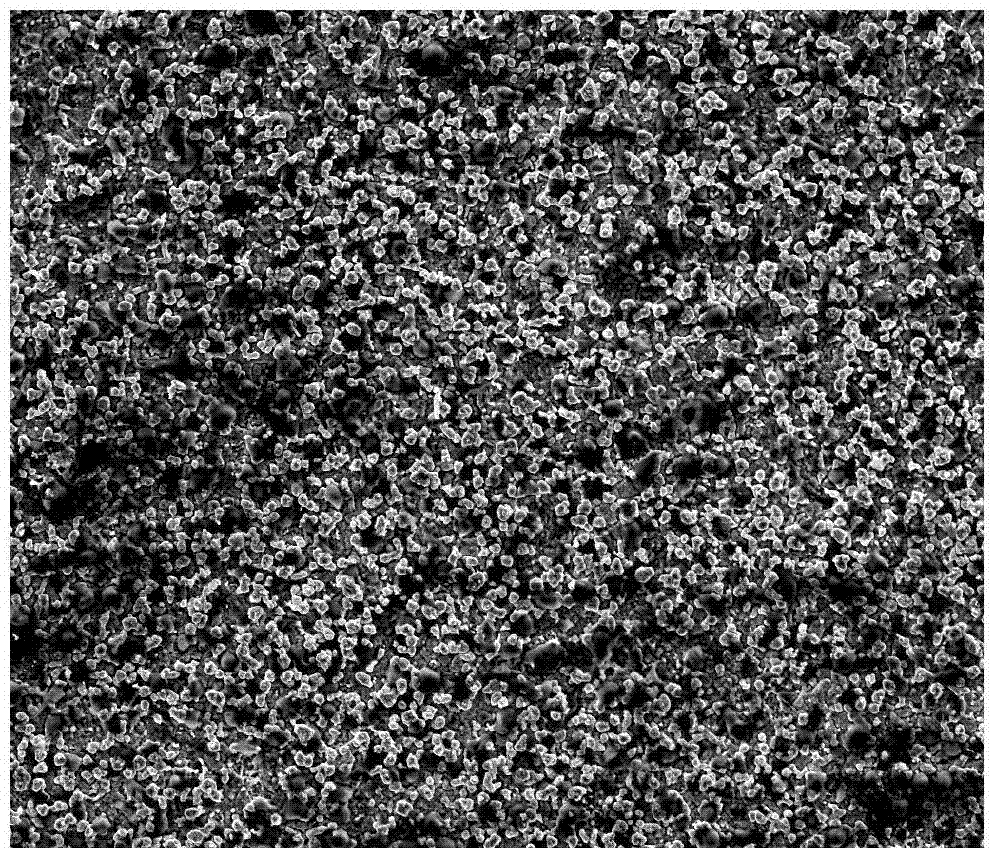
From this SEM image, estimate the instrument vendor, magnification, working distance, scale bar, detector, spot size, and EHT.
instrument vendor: FEI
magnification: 1 K X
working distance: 14.1 mm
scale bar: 100000 nm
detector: ETD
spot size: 5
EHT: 20 kV